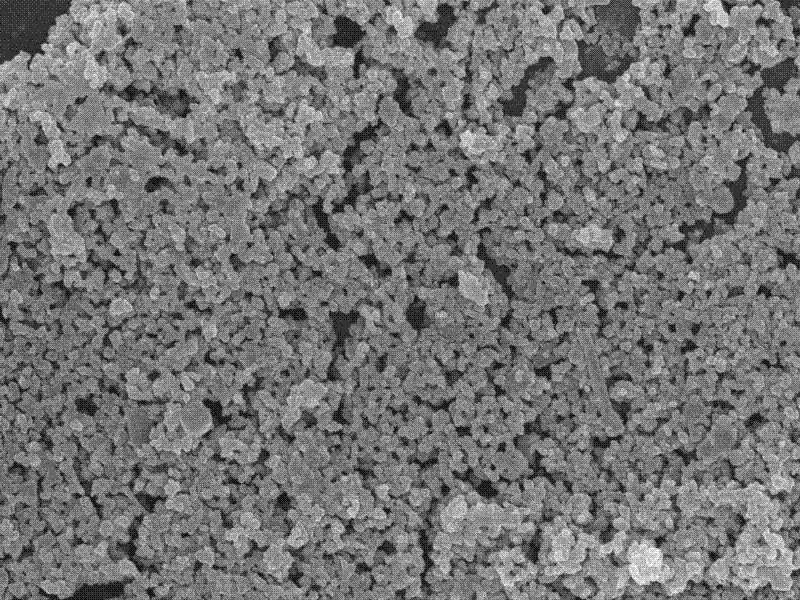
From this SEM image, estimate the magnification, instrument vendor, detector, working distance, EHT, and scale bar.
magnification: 10 K X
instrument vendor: JEOL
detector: SEI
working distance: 14 mm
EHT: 15 kV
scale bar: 1000 nm